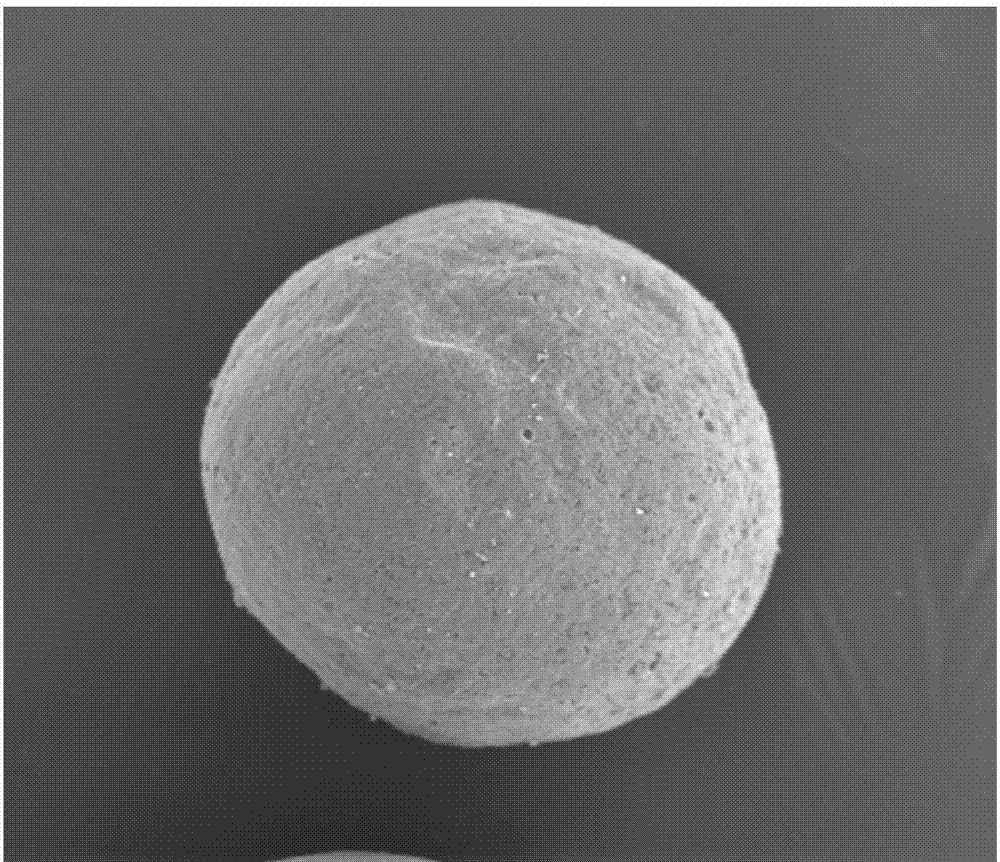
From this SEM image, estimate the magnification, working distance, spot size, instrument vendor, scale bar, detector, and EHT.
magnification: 0.3 K X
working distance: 10.3 mm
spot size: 6.5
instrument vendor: FEI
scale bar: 100000 nm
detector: ETD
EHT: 12.5 kV